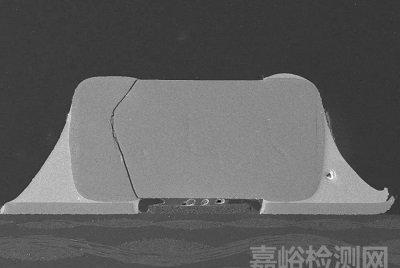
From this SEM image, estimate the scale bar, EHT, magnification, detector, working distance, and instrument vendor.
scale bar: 1000000 nm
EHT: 10 kV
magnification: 0.05 K X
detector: LM(L)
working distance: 15.4 mm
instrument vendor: Hitachi